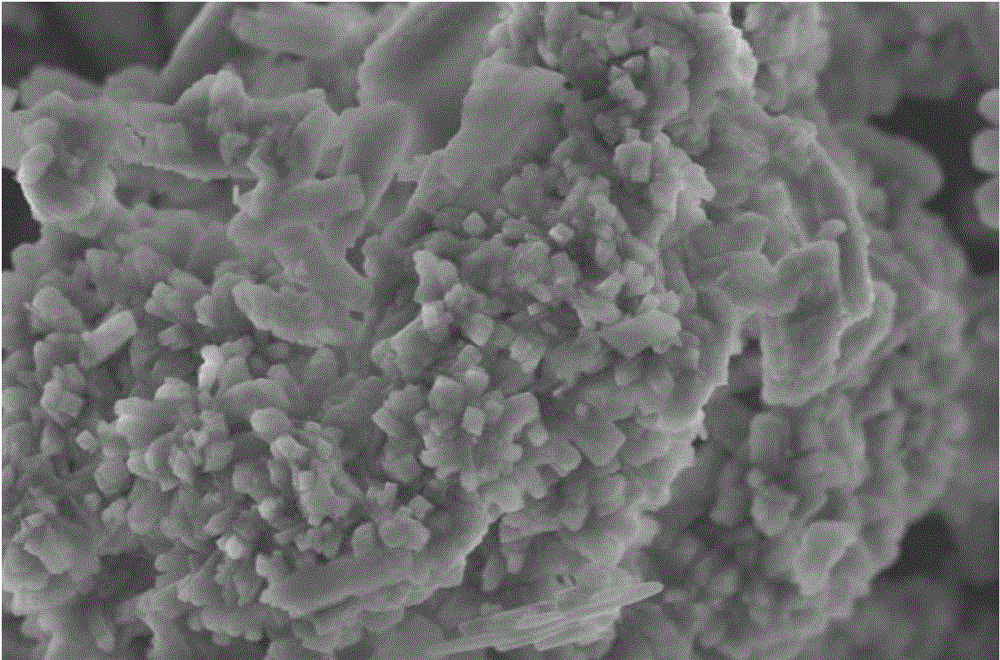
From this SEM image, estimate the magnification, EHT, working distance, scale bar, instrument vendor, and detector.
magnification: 12 K X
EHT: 5 kV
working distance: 8.4 mm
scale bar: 4000 nm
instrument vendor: Hitachi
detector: SE(M)